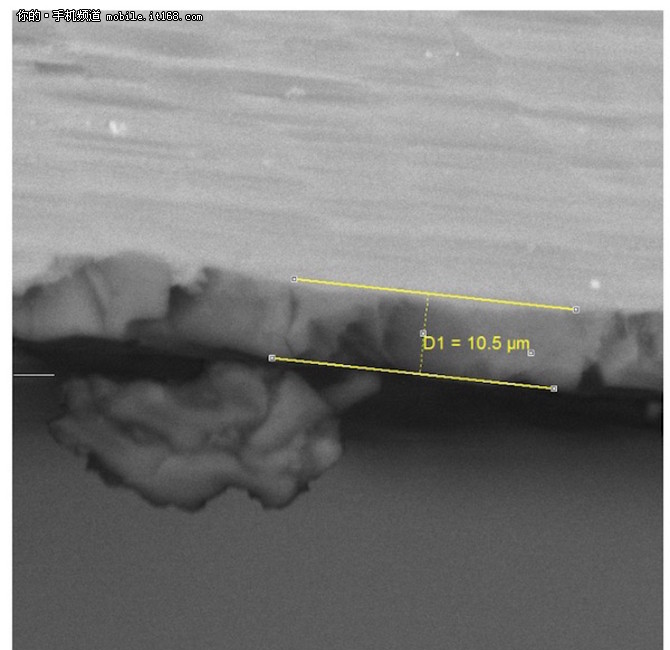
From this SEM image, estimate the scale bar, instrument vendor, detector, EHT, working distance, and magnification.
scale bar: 20000 nm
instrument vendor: Tescan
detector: BSE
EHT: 25 kV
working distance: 25.94 mm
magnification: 2.34 K X